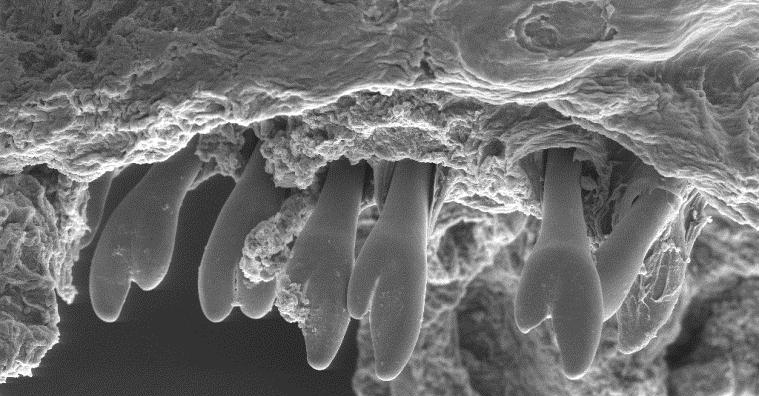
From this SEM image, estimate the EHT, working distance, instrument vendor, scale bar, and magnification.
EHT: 18 kV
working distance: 10 mm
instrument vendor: other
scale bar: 100000 nm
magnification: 0.165 K X